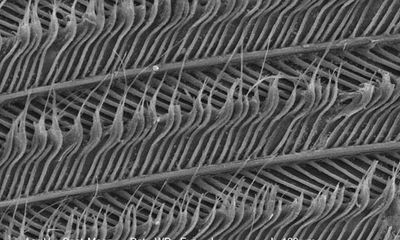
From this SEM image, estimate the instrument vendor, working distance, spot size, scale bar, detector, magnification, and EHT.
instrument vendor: FEI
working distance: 7.3 mm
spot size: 3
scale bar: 100000 nm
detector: SE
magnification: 0.186 K X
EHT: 30 kV